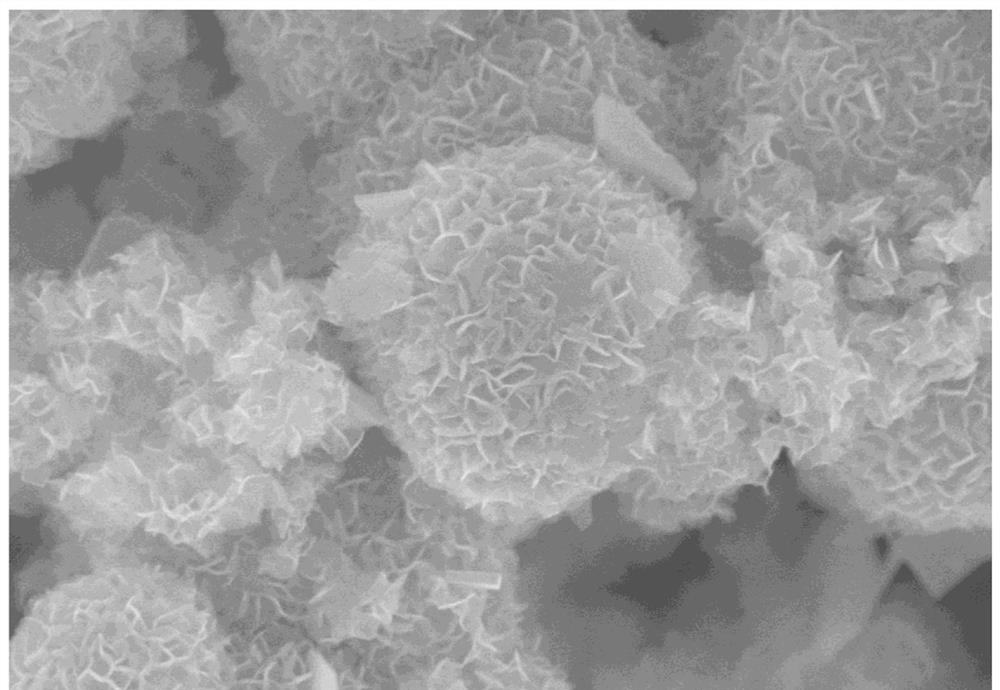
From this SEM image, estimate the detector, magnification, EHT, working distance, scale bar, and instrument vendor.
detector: SE(U)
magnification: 35 K X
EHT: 10 kV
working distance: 9.4 mm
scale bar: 1000 nm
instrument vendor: Hitachi